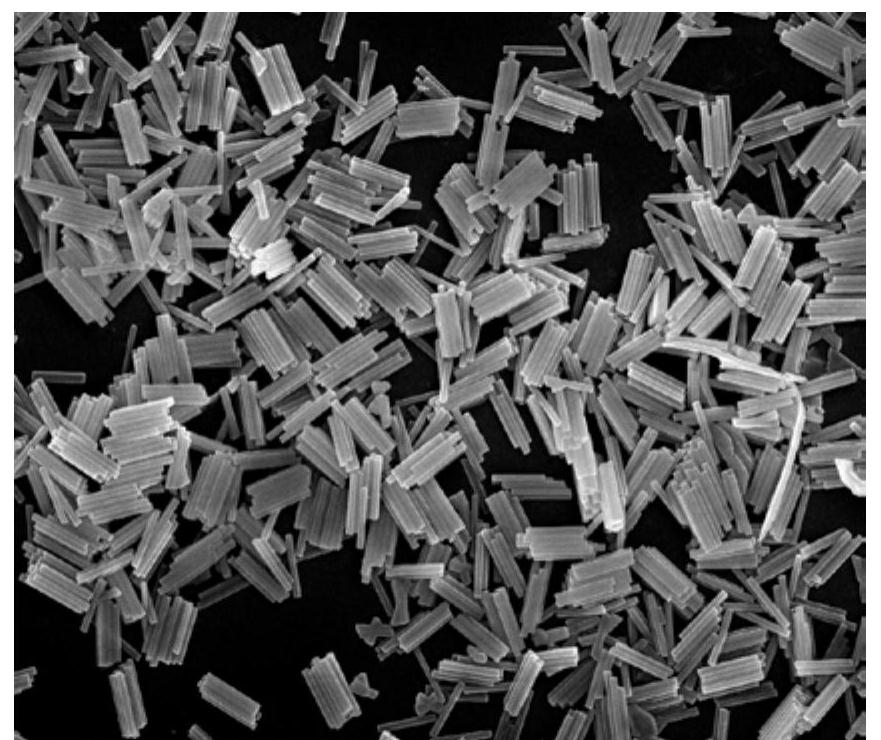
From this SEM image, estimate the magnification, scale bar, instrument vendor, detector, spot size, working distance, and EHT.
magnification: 20 K X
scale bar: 3000 nm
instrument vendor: FEI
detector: TLD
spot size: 3.5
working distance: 5.9 mm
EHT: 15 kV